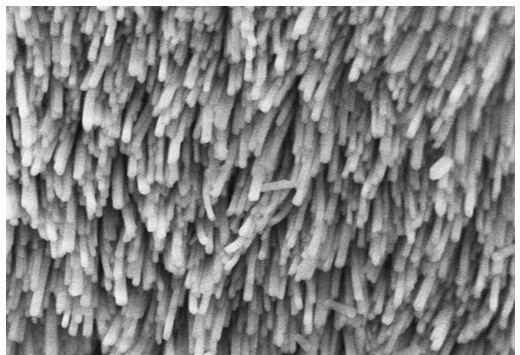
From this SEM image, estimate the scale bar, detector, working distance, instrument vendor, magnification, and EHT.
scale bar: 1000 nm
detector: SE(U)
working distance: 3.9 mm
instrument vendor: Hitachi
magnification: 50 K X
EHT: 3 kV